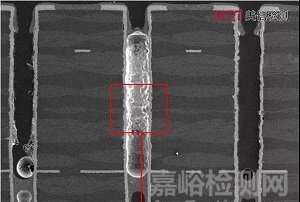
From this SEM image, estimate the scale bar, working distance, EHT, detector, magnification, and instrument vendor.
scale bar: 500000 nm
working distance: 10.2 mm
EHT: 15 kV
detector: SE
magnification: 0.06 K X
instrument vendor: Hitachi